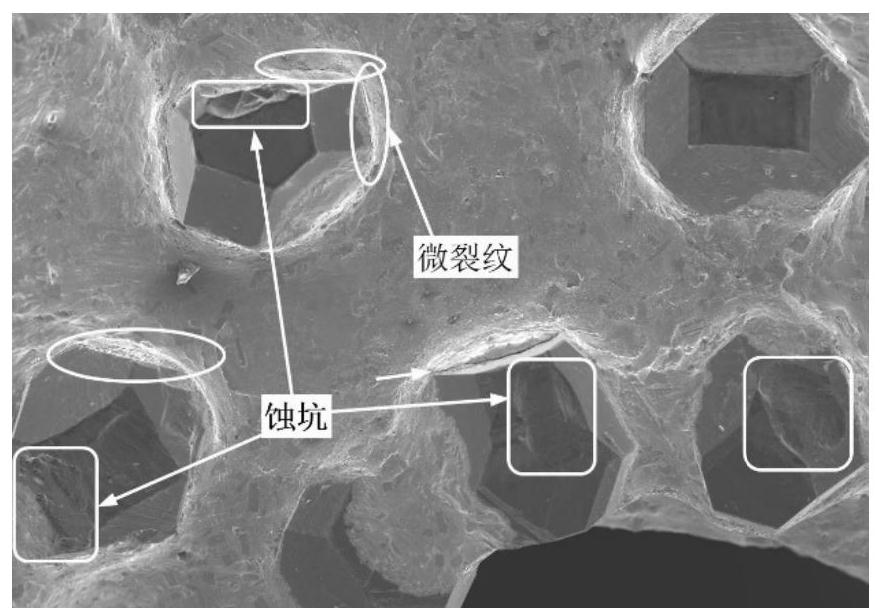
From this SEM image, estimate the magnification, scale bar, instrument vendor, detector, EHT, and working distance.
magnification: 0.06 K X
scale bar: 500000 nm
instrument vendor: Hitachi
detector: SE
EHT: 15 kV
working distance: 8.4 mm